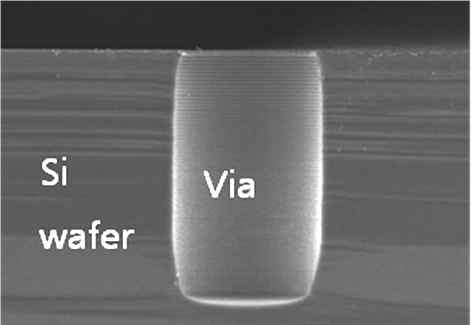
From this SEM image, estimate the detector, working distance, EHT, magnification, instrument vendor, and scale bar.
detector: SE(M)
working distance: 6.3 mm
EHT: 15 kV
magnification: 1 K X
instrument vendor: Hitachi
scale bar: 50000 nm